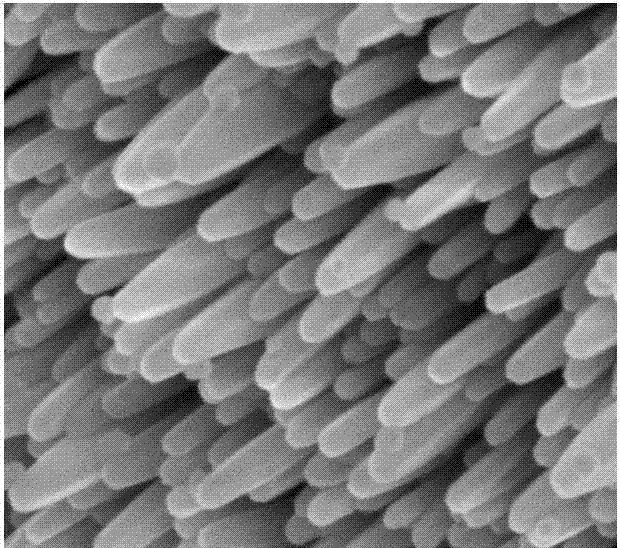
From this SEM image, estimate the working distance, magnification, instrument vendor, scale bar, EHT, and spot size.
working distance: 4.9 mm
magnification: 160 K X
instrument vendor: FEI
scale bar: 500 nm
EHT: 10 kV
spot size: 2.5